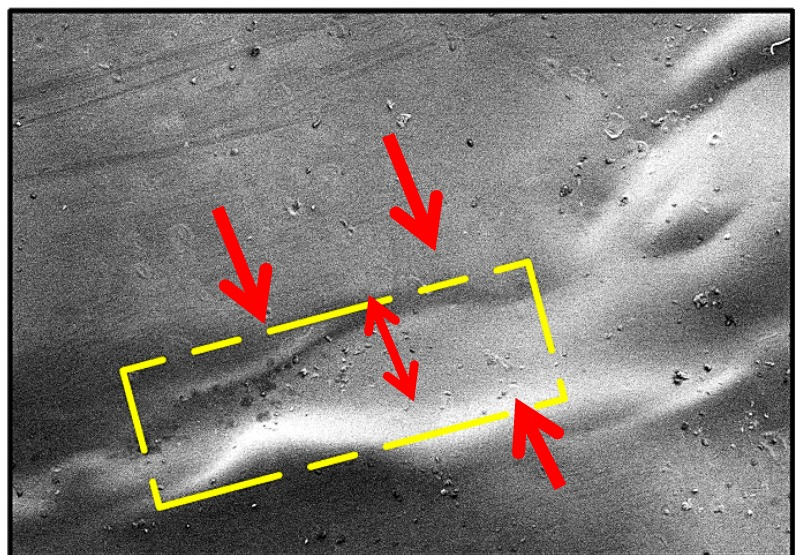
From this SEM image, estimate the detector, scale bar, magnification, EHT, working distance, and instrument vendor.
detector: LM(UL)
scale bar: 1000000 nm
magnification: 0.03 K X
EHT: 5 kV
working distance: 10.8 mm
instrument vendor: Hitachi